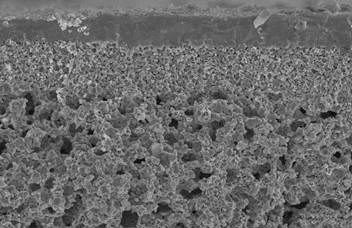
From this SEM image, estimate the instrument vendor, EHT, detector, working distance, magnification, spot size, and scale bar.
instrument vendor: FEI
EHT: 15 kV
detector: SE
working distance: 13.2 mm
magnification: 1.25 K X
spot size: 3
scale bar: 20000 nm